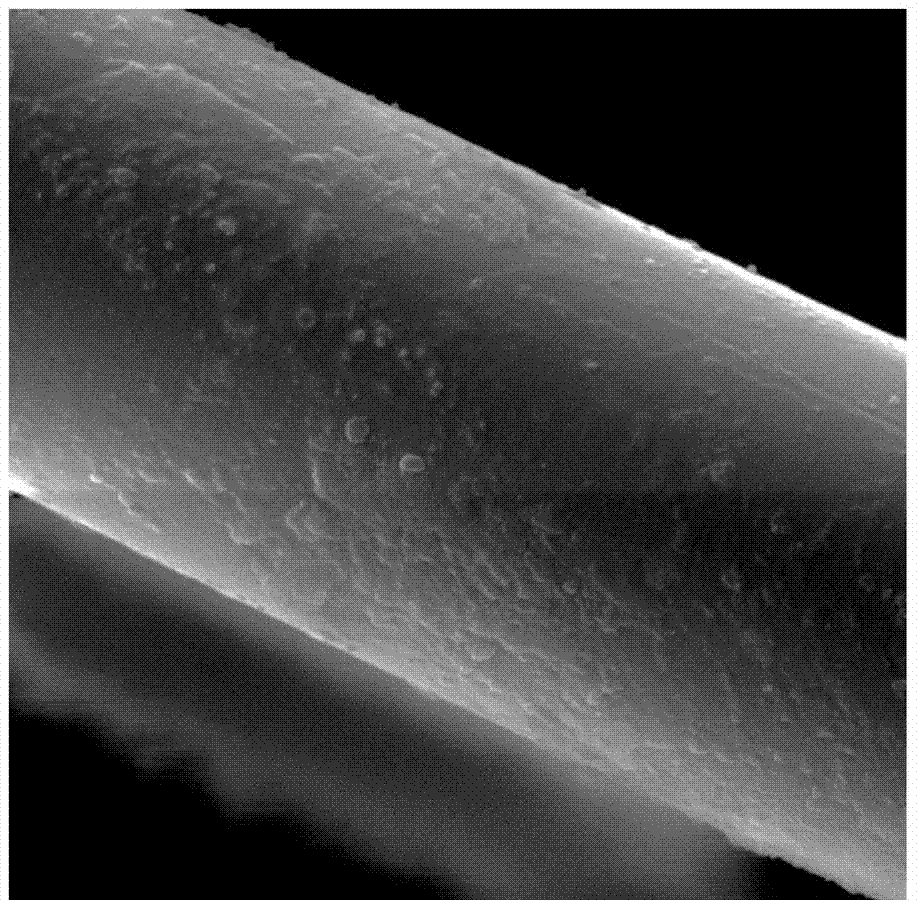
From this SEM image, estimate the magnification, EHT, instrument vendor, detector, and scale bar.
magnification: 5 K X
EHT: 20 kV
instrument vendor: Tescan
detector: SE Detector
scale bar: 10000 nm